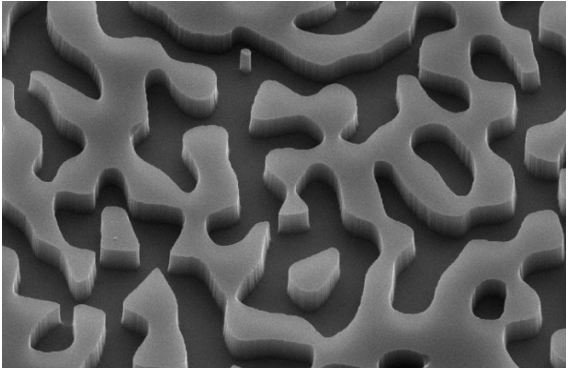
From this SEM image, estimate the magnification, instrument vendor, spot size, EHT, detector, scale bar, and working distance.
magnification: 20 K X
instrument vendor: FEI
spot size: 3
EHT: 10 kV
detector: SE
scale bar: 2000 nm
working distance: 6 mm